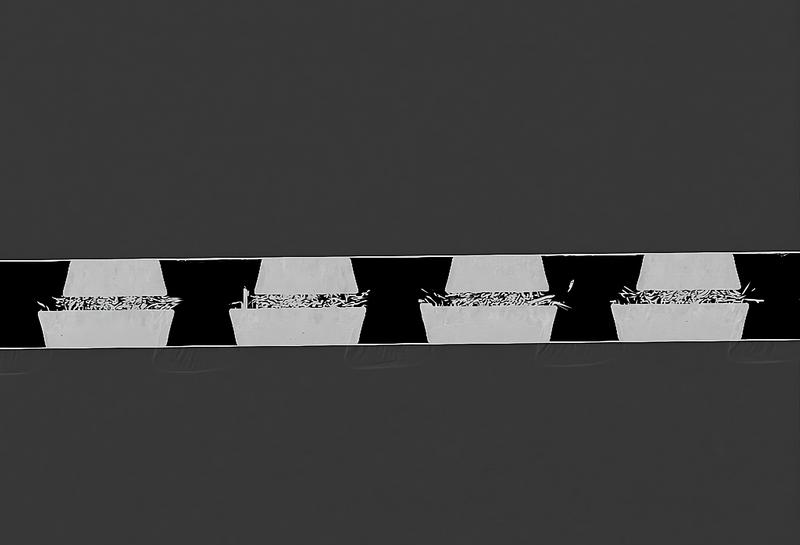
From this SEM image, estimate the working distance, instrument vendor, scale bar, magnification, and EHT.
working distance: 8 mm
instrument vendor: Zeiss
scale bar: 20000 nm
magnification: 0.5 K X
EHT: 10 kV